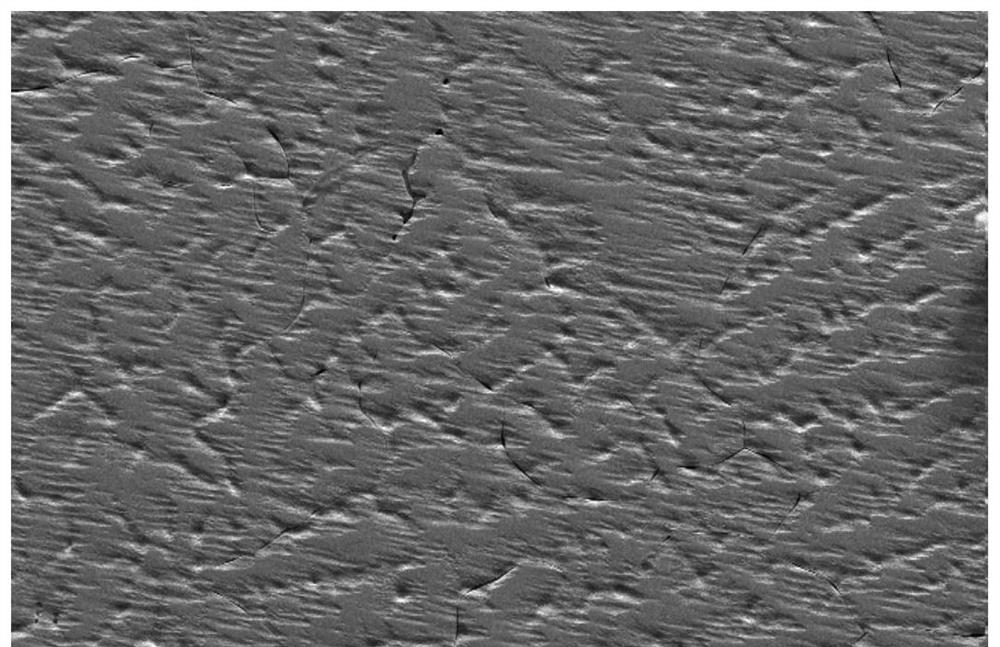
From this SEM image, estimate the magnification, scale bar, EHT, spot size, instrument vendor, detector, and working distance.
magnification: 0.5 K X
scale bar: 100000 nm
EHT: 20 kV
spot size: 4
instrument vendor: FEI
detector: SE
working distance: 5.2 mm